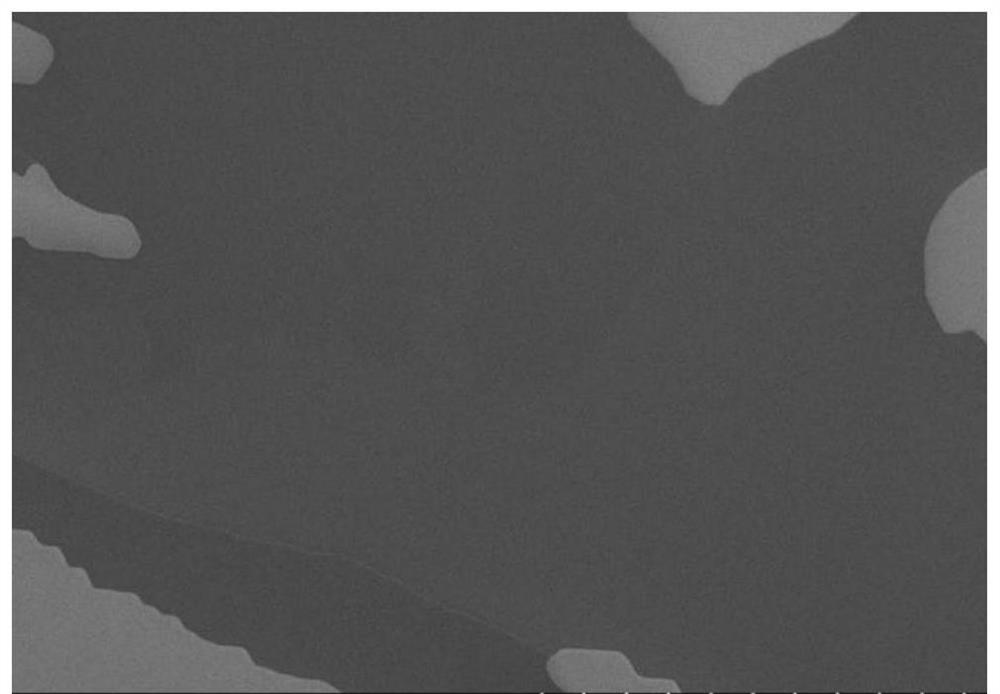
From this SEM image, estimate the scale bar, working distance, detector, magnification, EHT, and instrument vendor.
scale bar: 100000 nm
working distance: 5.2 mm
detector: SE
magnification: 0.55 K X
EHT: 15 kV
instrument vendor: Hitachi